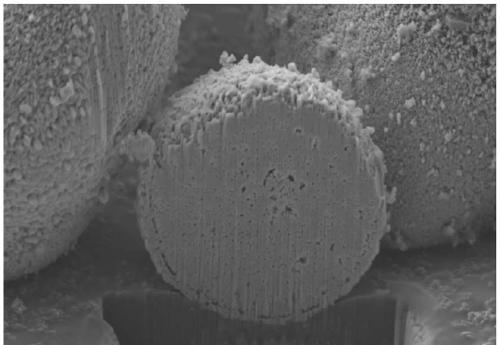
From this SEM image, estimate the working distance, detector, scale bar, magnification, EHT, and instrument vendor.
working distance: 5.1 mm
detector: SE2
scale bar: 1000 nm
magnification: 5 K X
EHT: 5 kV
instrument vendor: Zeiss